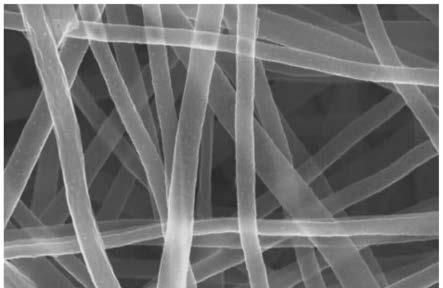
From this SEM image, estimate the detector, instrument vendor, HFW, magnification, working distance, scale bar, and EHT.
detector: ETD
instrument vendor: FEI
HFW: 6.91 µm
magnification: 60 K X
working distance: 12.5 mm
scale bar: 2000 nm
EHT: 30 kV